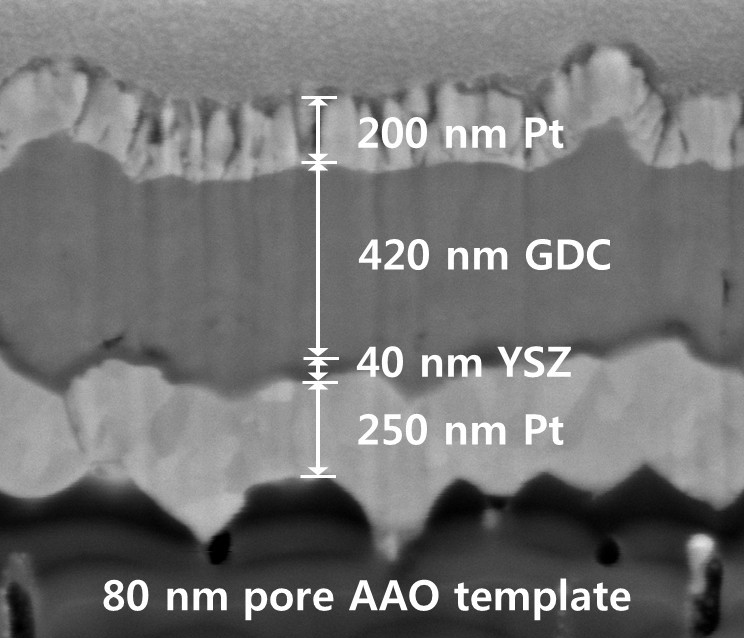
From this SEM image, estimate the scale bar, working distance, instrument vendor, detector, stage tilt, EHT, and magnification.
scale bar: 400 nm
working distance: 10 mm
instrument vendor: FEI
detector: ETD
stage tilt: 52°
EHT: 5 kV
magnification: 100 K X